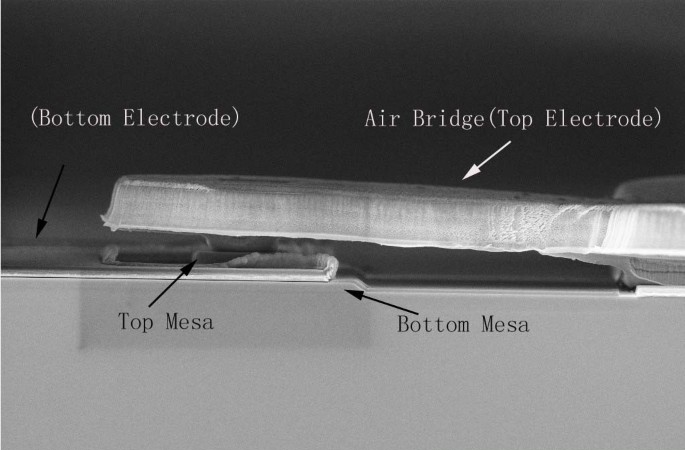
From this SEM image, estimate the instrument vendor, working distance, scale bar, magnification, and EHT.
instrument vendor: Zeiss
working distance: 5 mm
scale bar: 2000 nm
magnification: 5 K X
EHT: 5 kV